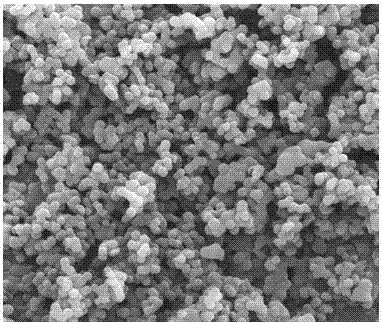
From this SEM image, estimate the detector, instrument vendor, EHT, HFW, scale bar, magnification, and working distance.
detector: TLD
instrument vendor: FEI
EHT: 10 kV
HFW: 12 µm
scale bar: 3000 nm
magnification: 20 K X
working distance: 5.3 mm